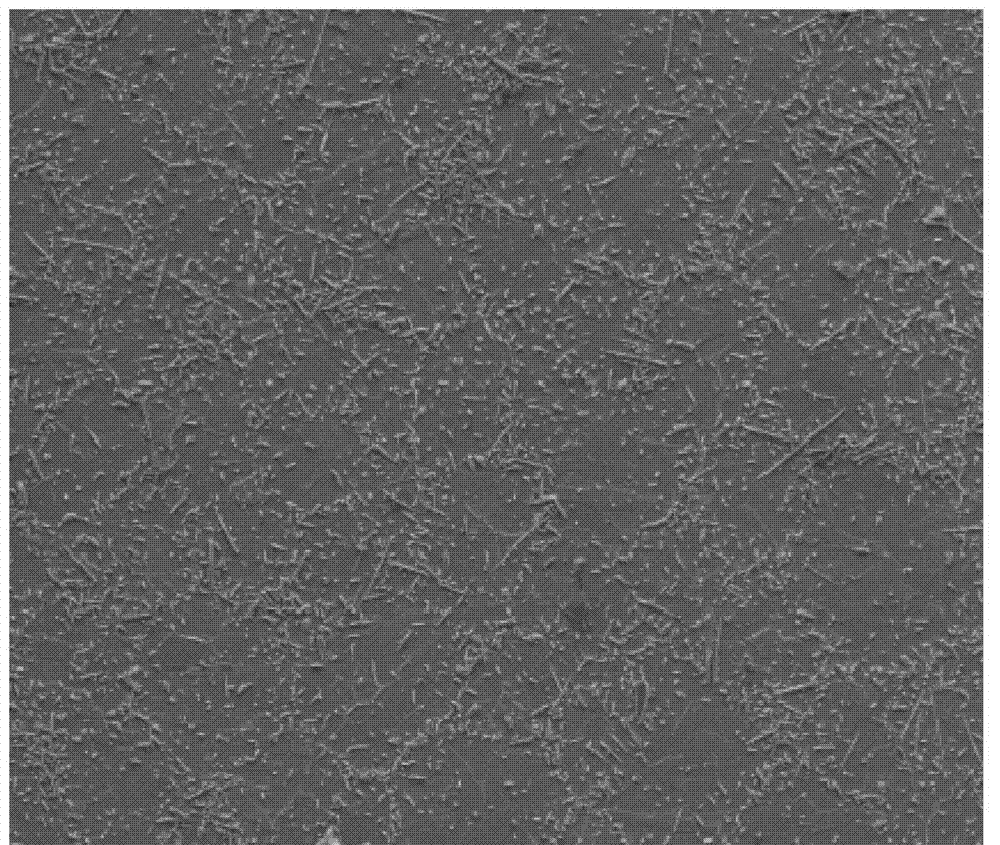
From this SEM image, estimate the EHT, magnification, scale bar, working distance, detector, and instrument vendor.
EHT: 20 kV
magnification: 0.3 K X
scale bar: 400000 nm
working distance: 17.8 mm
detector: SE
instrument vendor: FEI